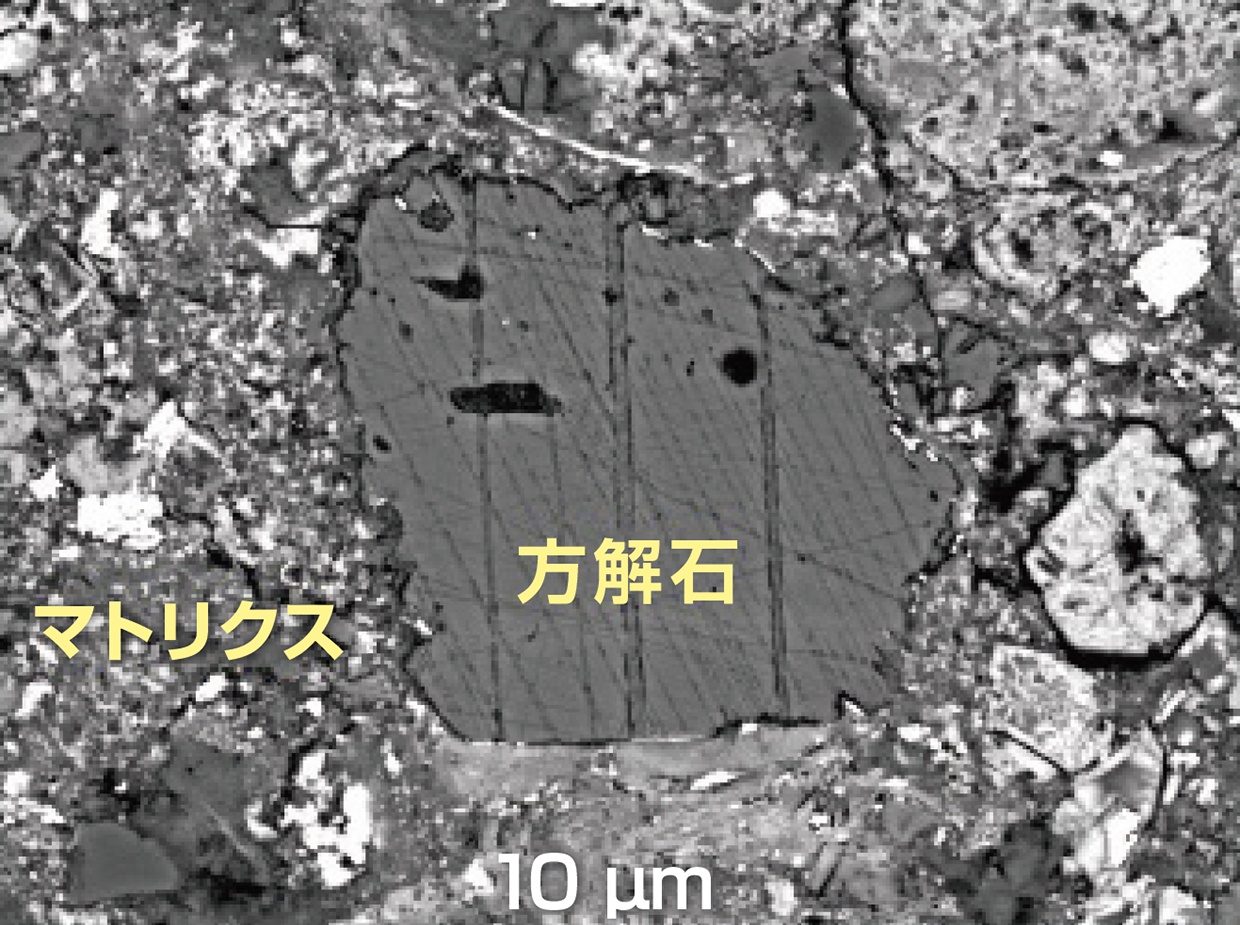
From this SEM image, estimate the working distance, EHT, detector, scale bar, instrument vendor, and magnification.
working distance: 10 mm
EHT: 15 kV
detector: BEI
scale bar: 10000 nm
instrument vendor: JEOL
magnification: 1.5 K X